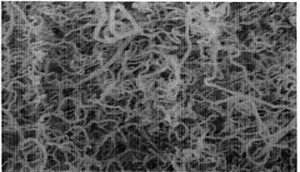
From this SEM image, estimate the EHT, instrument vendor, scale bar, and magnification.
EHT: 10 kV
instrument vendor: Hitachi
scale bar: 3000 nm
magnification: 15 K X